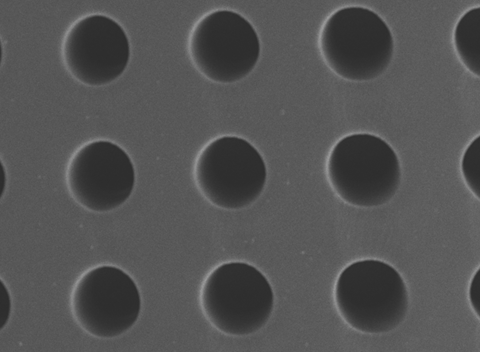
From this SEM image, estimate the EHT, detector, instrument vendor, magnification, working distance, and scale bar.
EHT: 5 kV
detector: LED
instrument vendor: JEOL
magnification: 3 K X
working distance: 8 mm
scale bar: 1000 nm